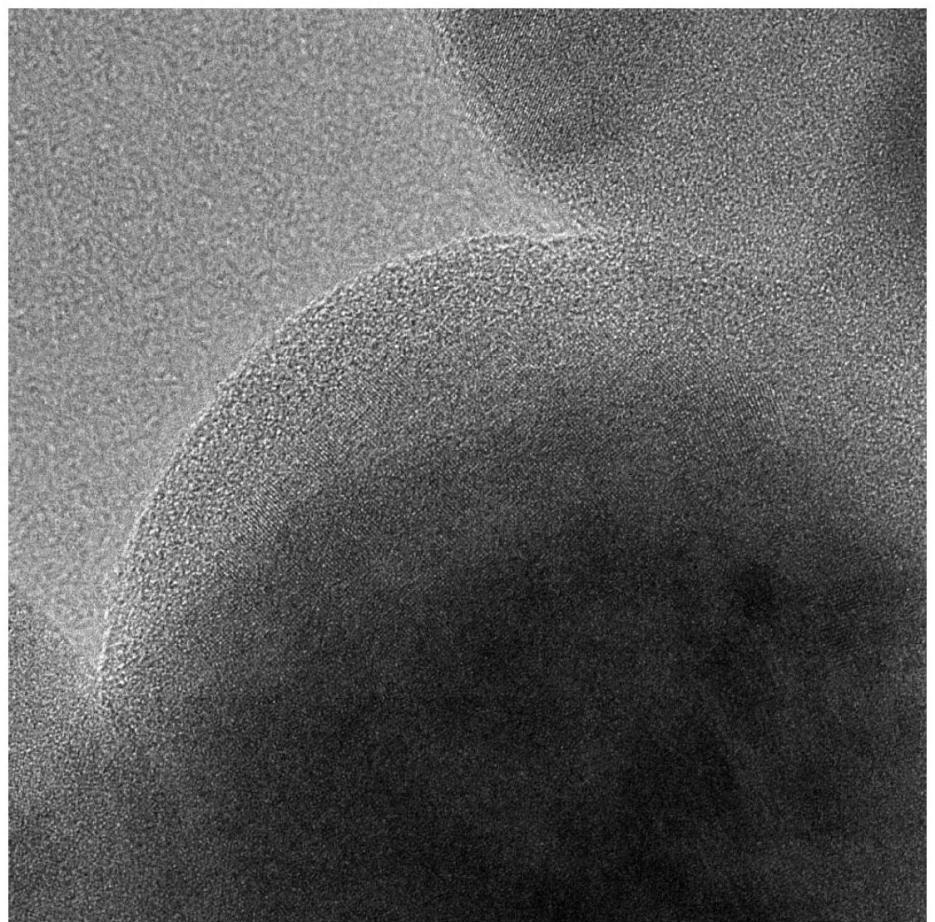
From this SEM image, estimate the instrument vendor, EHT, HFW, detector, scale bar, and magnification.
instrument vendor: Thermo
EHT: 200 kV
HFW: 0.06625 µm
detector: Ceta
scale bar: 10 nm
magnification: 440 K X